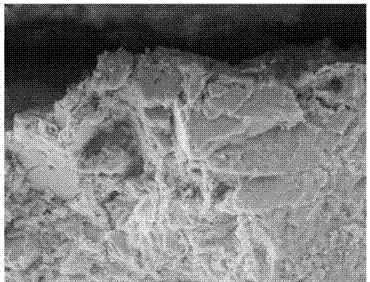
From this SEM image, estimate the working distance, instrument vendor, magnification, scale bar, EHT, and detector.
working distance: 9 mm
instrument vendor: Zeiss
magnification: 2 K X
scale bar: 3000 nm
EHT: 8 kV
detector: SE1